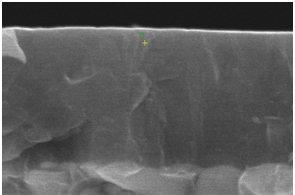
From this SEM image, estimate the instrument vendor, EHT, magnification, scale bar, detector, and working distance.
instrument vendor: Tescan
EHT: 15 kV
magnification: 50 K X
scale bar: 500 nm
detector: SE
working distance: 11.7 mm